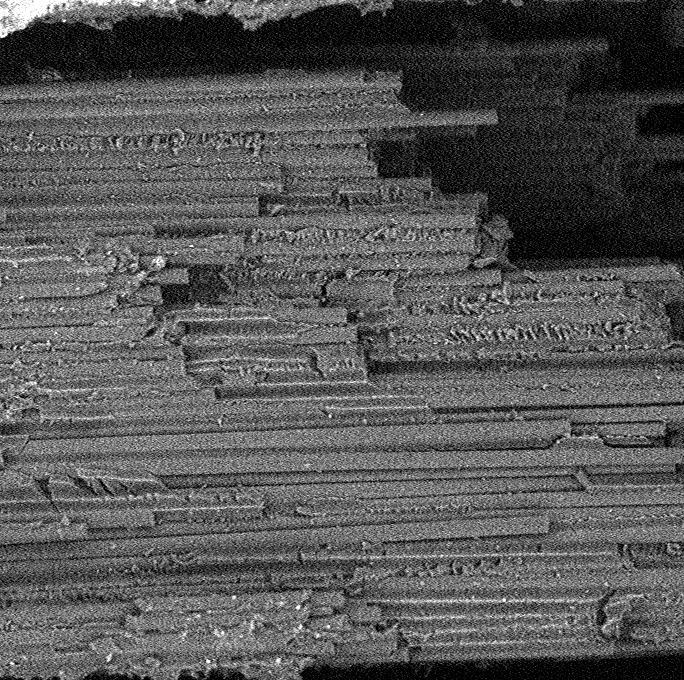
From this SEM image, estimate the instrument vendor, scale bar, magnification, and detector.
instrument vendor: other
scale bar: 80000 nm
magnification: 0.89 K X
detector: BSD Full